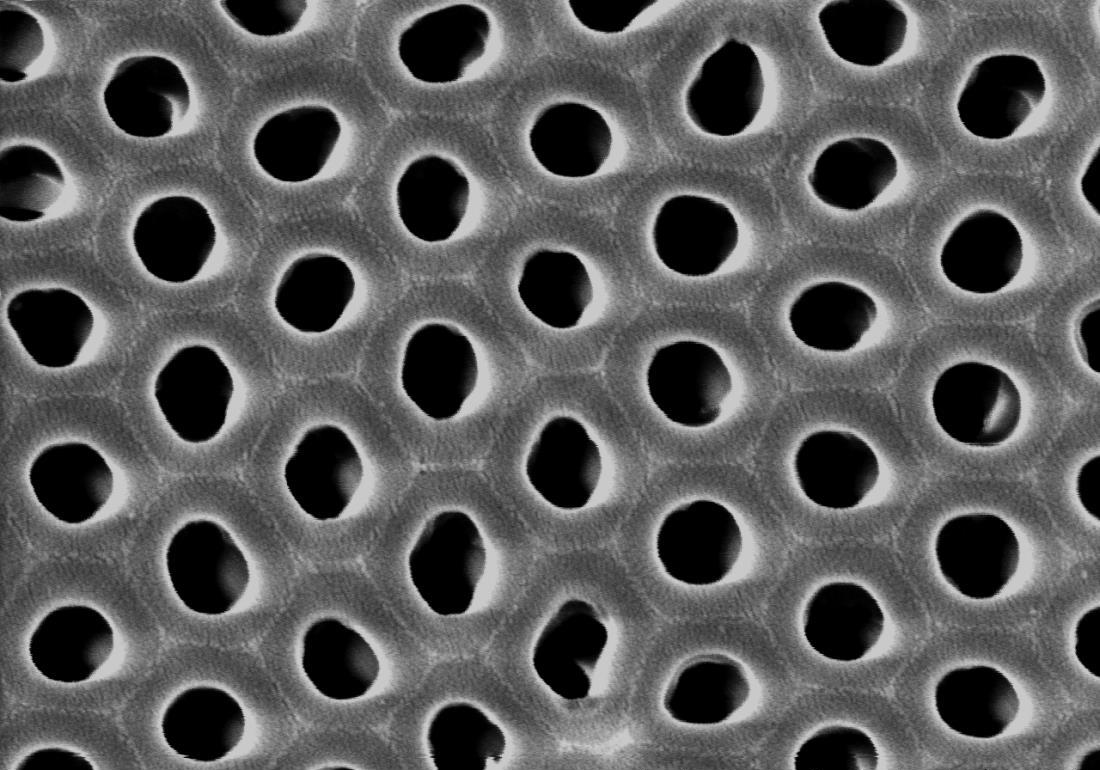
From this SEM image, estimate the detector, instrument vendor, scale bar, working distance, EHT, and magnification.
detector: SE(M)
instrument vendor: Hitachi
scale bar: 1000 nm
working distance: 12.9 mm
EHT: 20 kV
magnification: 50 K X